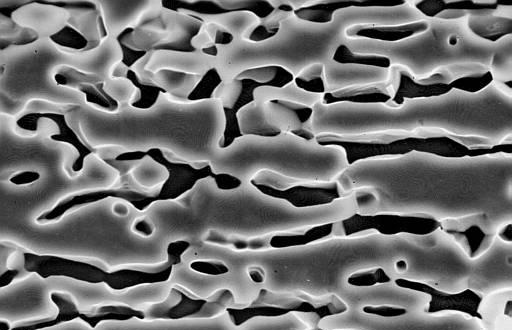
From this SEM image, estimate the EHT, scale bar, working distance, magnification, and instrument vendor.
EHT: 20 kV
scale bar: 10000 nm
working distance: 14 mm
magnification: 3.5 K X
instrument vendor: JEOL